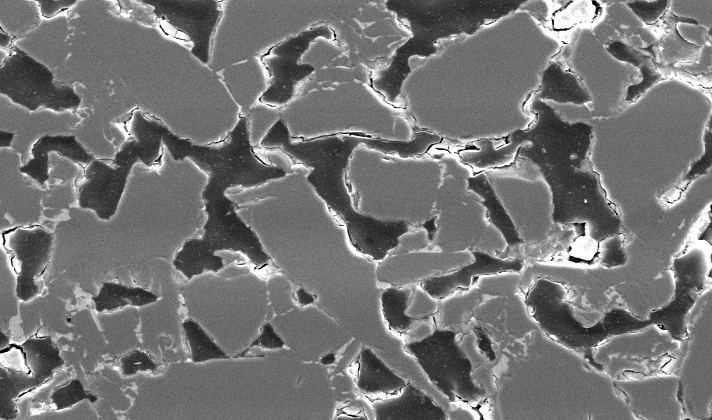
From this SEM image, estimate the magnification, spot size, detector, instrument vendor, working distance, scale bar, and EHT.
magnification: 0.5 K X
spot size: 3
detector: SE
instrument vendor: FEI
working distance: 4.9 mm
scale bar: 50000 nm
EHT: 20 kV